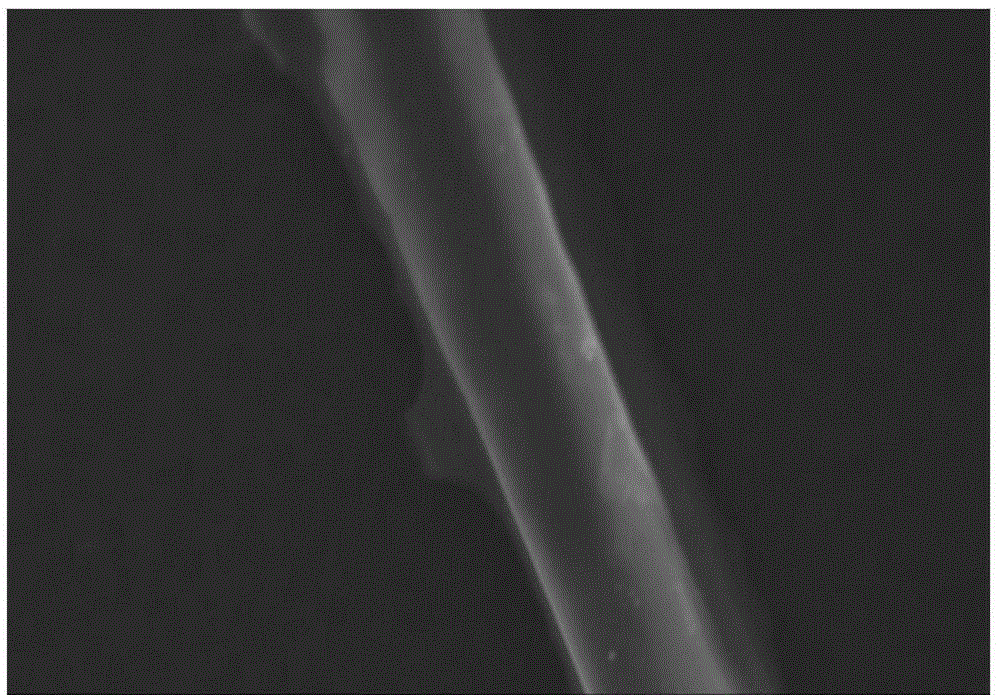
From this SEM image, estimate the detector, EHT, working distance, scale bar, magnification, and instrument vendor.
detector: SE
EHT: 15 kV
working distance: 4.5 mm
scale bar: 30000 nm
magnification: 1.5 K X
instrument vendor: Hitachi